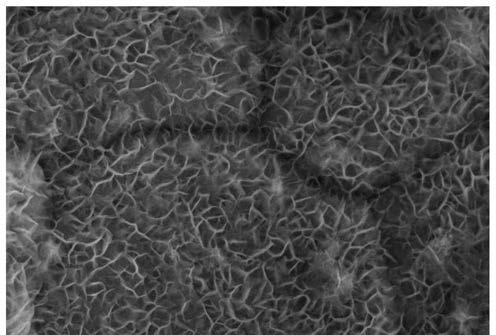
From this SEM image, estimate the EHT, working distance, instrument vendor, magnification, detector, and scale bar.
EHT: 10 kV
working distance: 7.5 mm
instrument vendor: Zeiss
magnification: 20 K X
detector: InLens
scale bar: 200 nm